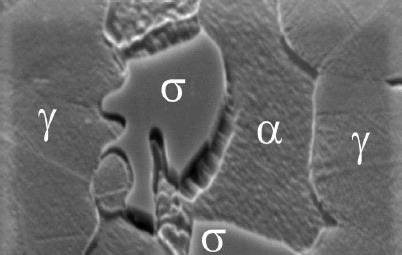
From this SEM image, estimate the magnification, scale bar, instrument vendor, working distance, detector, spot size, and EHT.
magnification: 6.226 K X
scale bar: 10000 nm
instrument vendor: FEI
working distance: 15.1 mm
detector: SE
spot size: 5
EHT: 25 kV